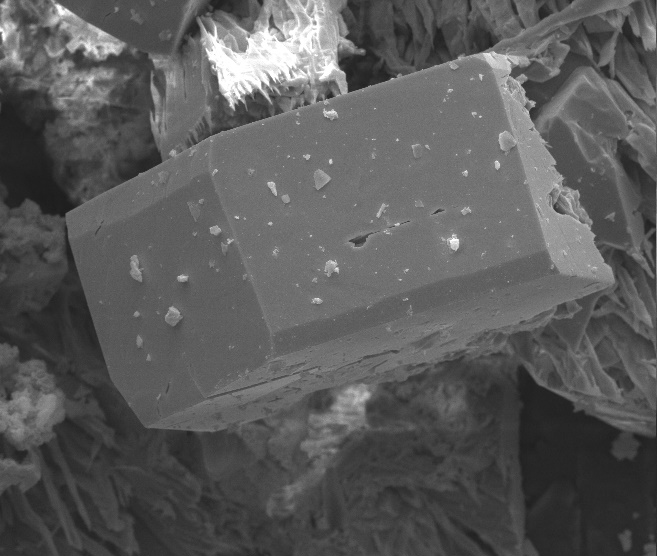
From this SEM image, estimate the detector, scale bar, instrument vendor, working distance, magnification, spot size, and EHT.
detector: ETD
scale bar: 50000 nm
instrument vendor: FEI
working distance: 21.4 mm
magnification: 1.138 K X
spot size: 4.5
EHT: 25 kV